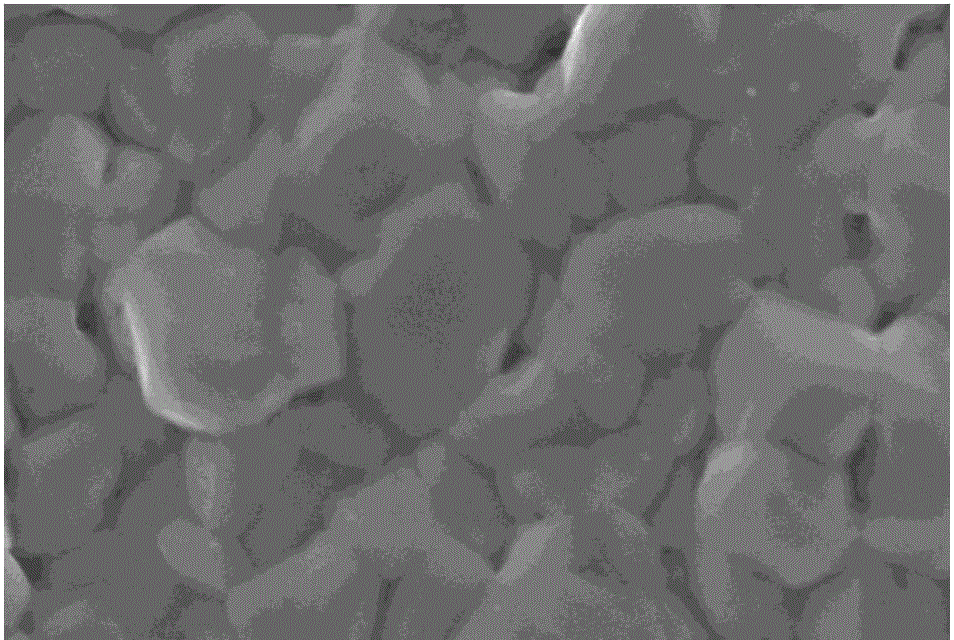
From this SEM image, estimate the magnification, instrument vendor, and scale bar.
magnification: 10 K X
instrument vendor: JEOL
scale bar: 1000 nm